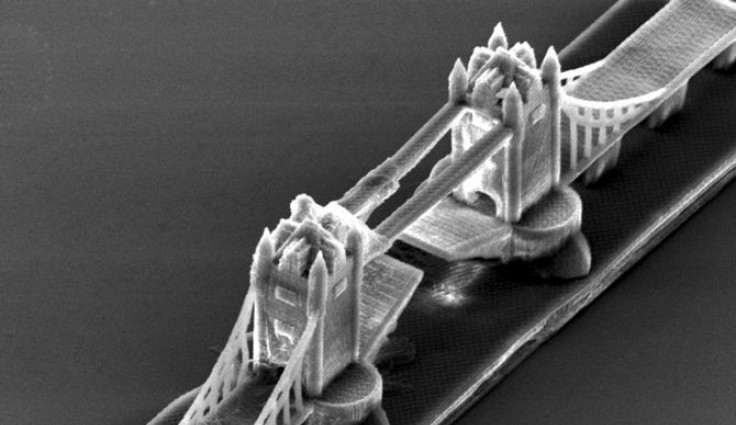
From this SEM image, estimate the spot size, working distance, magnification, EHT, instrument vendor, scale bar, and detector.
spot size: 4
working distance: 27.1 mm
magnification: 0.636 K X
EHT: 15 kV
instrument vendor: FEI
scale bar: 50000 nm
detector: SE